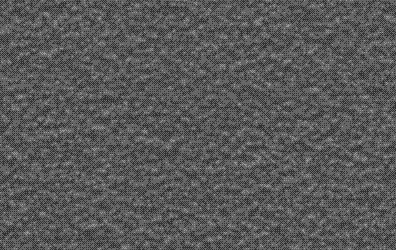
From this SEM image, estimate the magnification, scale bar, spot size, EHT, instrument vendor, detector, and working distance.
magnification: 50 K X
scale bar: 1000 nm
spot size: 3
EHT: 5 kV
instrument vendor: FEI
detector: SE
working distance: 6.9 mm